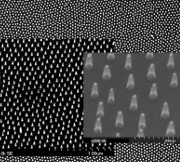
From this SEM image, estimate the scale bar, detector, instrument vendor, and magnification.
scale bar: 3000 nm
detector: SE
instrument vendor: Hitachi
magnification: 15 K X